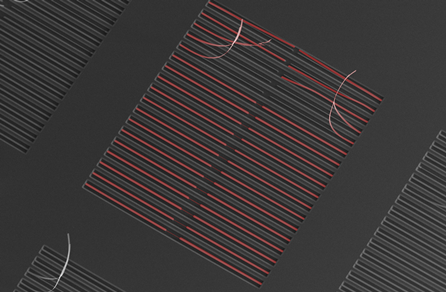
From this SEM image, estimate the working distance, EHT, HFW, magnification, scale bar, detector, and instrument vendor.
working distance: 5 mm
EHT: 10 kV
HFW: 3190 µm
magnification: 0.065 K X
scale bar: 500000 nm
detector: SE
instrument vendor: FEI